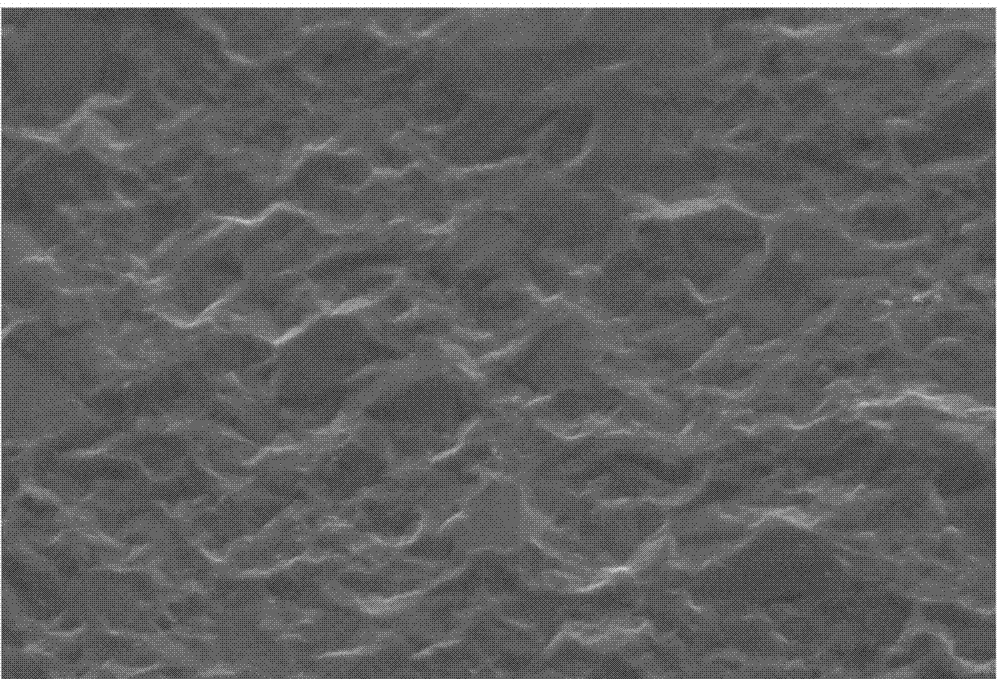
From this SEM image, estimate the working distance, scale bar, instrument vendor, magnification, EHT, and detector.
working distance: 18.8 mm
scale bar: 10000 nm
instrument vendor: Hitachi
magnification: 3 K X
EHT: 15 kV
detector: SE